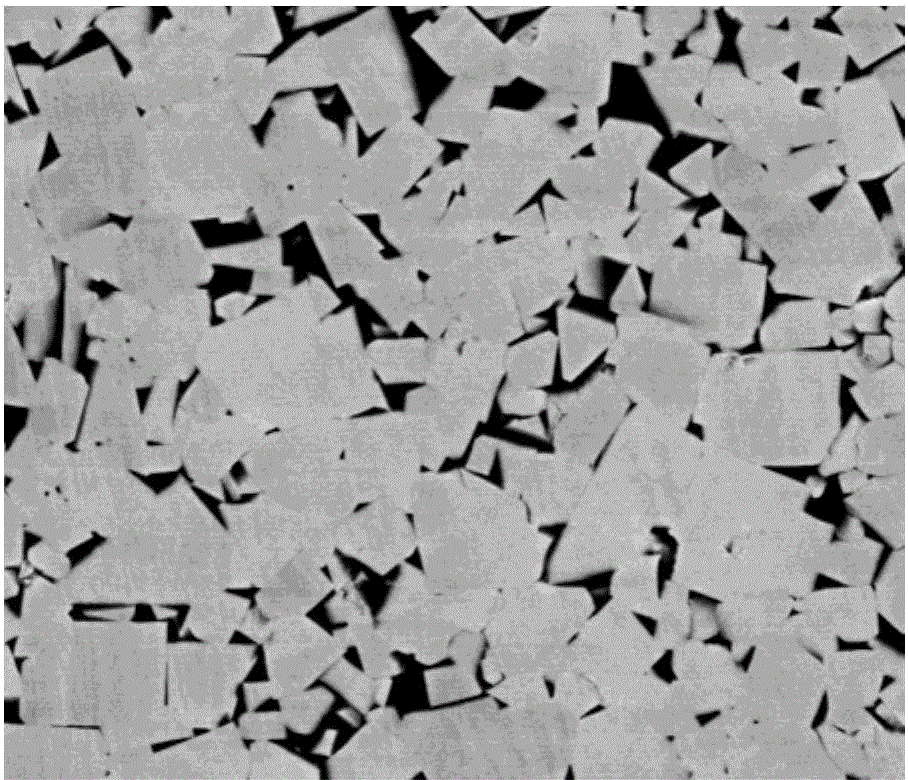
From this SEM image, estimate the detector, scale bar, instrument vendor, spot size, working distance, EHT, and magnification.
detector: BSED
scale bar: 10000 nm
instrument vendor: FEI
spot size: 4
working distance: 11.4 mm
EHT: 20 kV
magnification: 10 K X